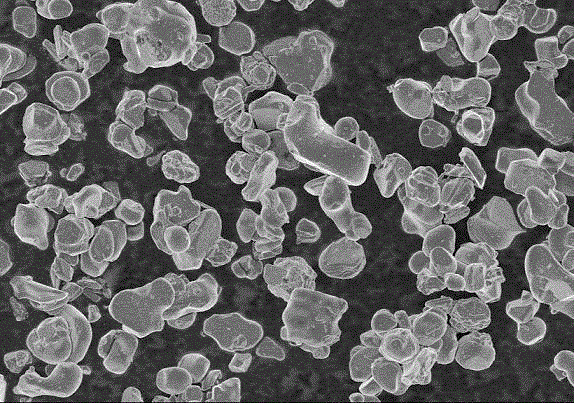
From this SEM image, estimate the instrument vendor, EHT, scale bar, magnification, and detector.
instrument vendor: Hitachi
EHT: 4 kV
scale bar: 50000 nm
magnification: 1 K X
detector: SE(M)